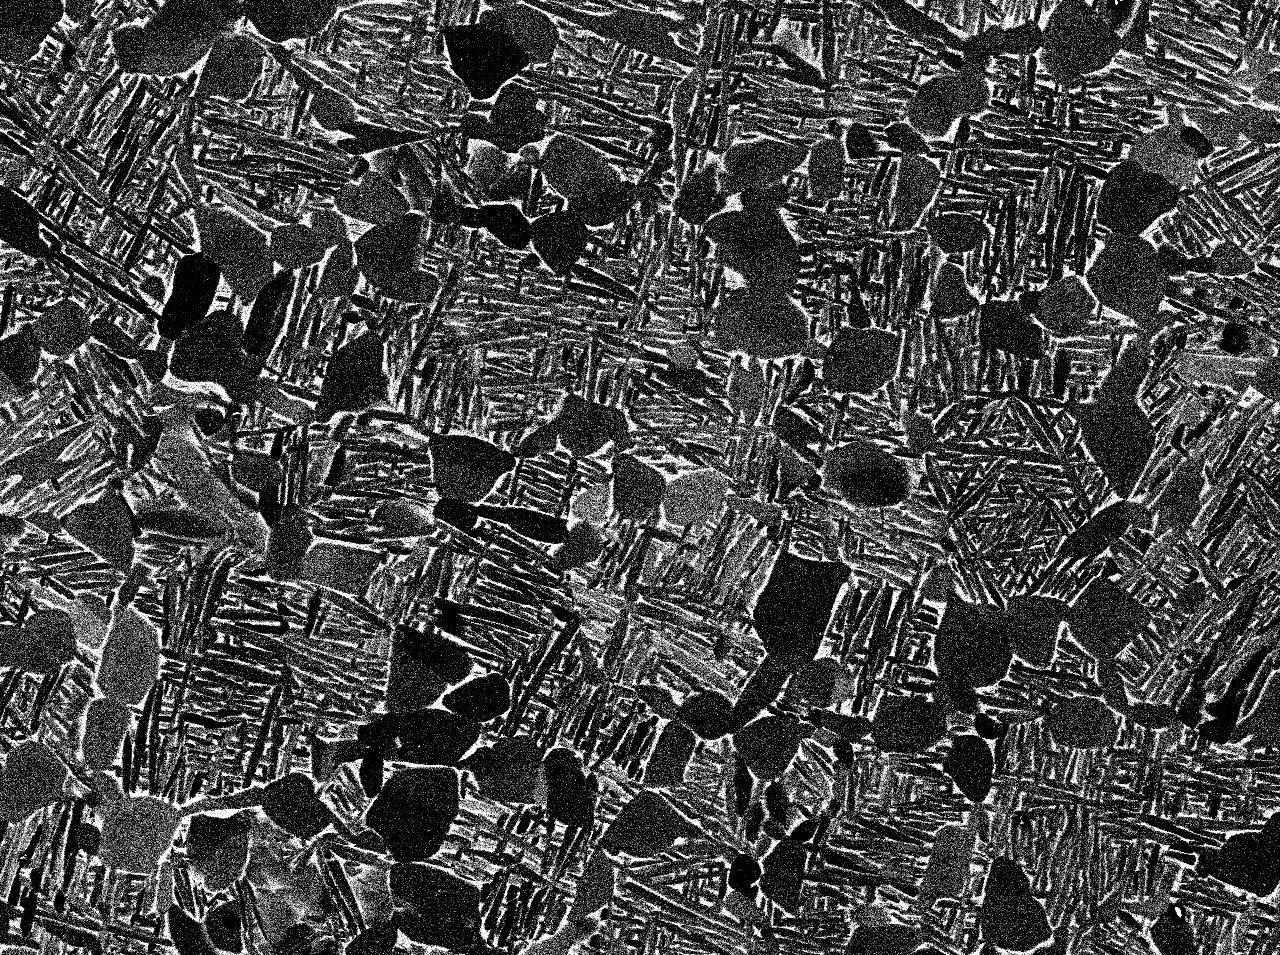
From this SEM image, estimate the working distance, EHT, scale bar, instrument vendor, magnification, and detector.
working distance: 10.1 mm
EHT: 15 kV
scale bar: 10000 nm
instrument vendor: JEOL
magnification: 1 K X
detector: COMPO